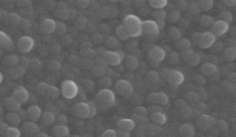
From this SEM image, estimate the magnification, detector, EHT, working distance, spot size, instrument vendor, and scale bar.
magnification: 22.996 K X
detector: GSE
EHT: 30 kV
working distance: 9.9 mm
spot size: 3.6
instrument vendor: FEI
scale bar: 1000 nm